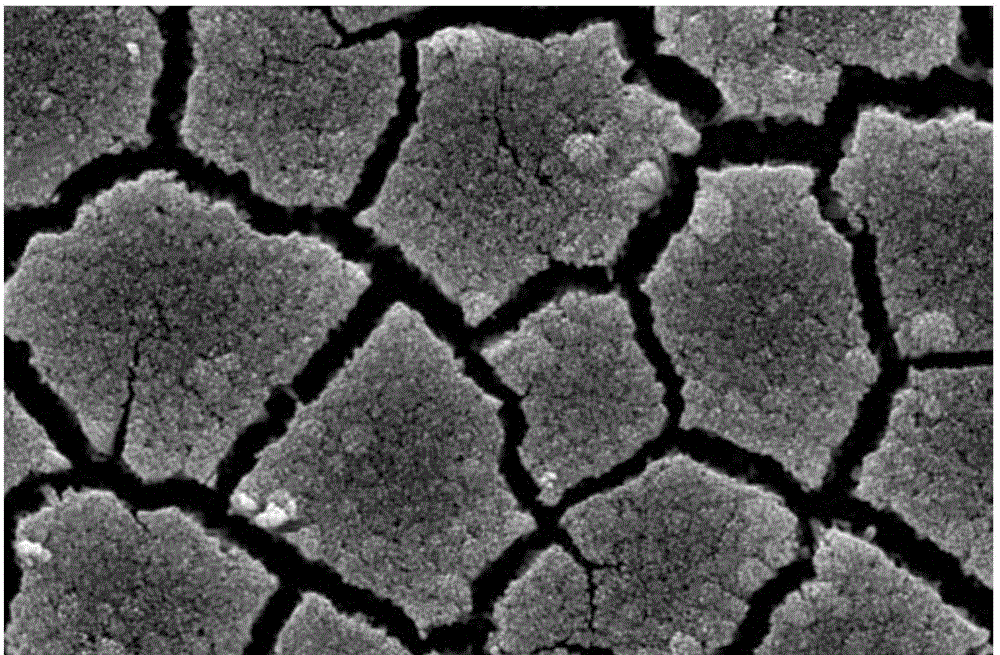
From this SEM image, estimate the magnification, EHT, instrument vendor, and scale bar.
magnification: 10 K X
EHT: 15 kV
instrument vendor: JEOL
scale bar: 1000 nm